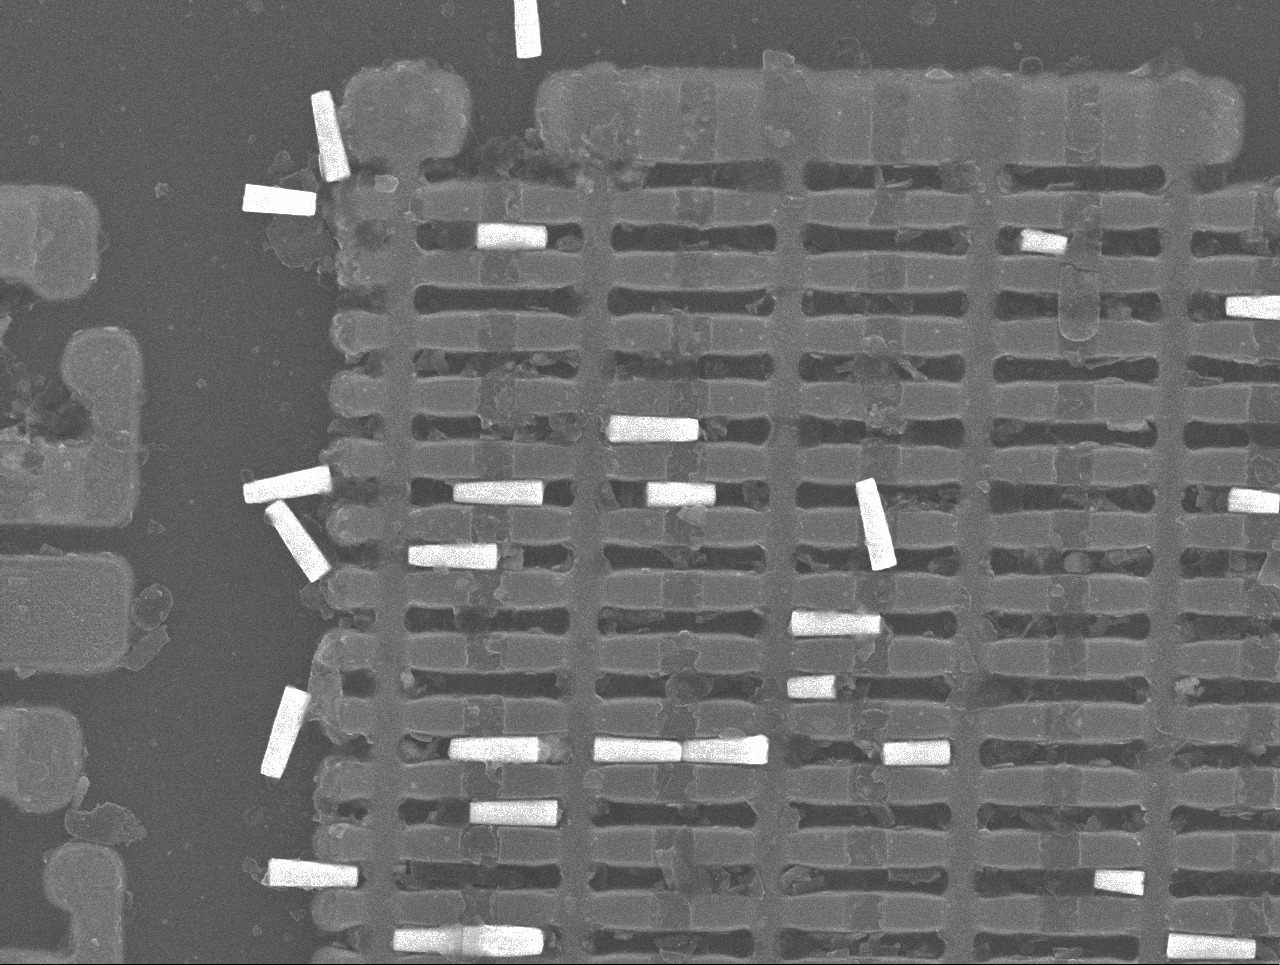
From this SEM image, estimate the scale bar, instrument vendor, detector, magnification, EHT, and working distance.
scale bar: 1000 nm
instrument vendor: JEOL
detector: SEI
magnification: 9 K X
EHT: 15 kV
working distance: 15 mm